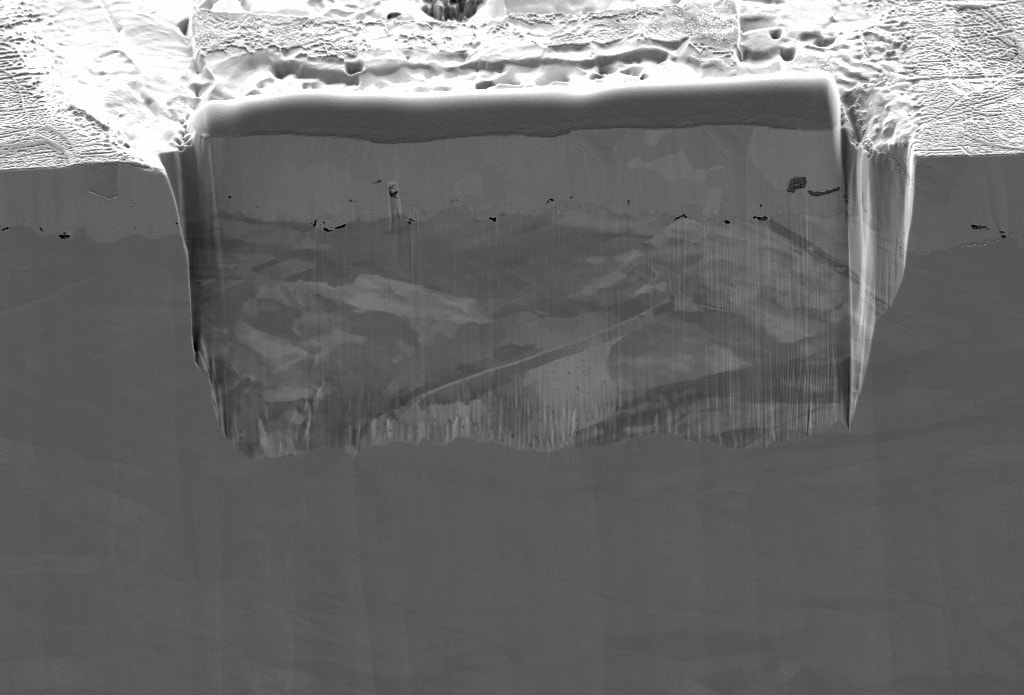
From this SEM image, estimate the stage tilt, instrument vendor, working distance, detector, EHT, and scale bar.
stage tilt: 54°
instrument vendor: Zeiss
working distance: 4.9 mm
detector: SESI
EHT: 2 kV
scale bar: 2000 nm